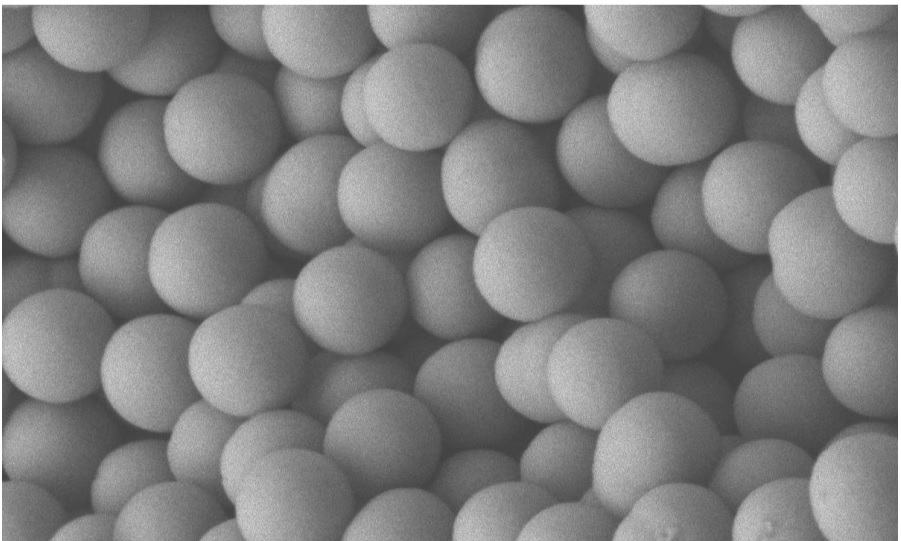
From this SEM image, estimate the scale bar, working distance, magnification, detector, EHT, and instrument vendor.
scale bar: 100 nm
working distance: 8.4 mm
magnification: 30 K X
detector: LEI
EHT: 5 kV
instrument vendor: JEOL